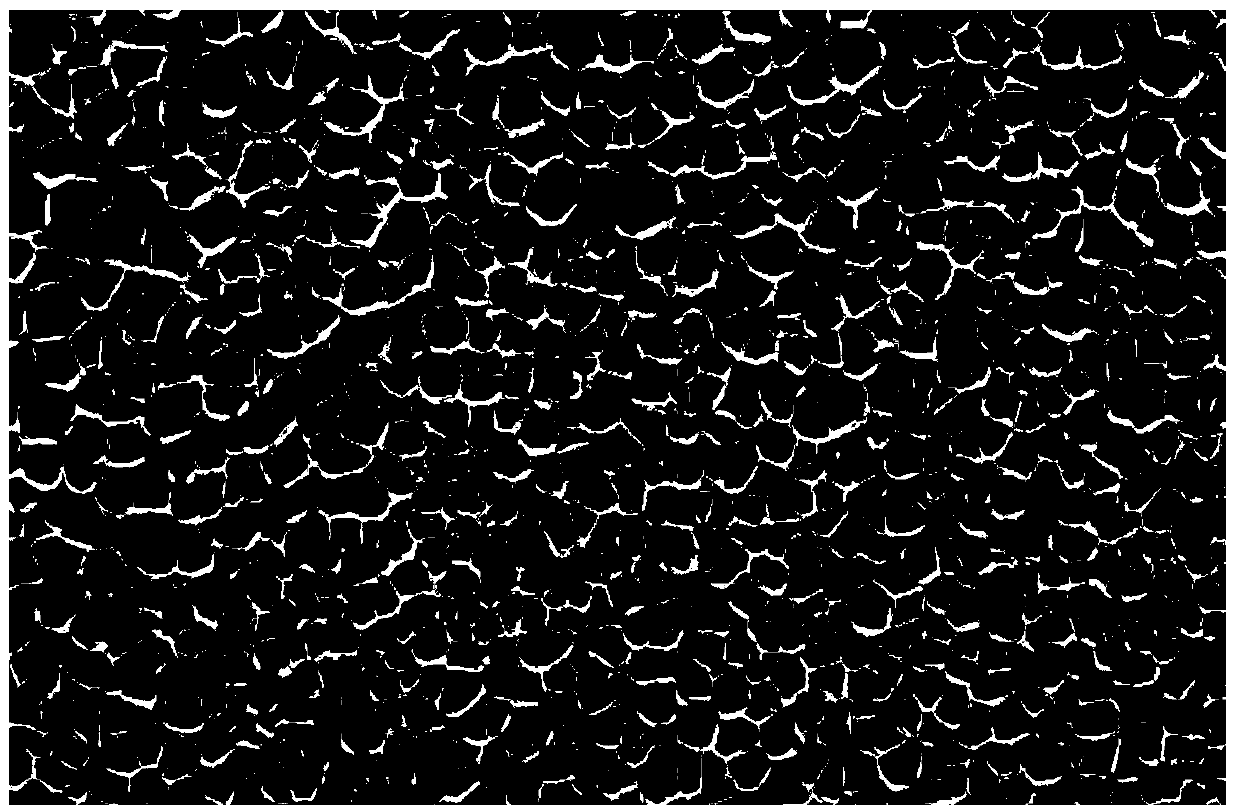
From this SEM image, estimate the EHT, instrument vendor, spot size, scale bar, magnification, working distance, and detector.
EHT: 15 kV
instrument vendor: FEI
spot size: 3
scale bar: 100000 nm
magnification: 0.2 K X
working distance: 7.5 mm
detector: SE